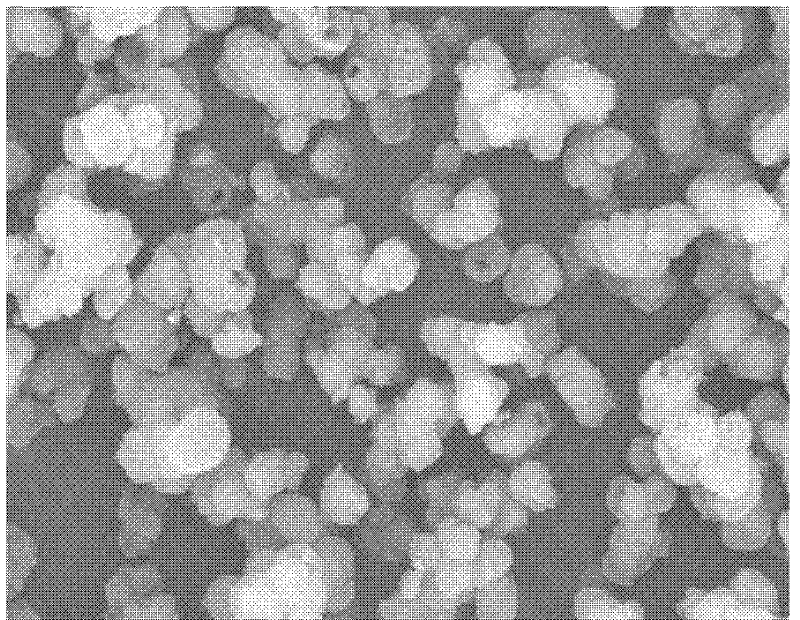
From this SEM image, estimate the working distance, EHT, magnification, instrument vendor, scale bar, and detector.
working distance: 12.6 mm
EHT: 15 kV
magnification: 2.5 K X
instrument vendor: Hitachi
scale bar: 20000 nm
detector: SE(M)